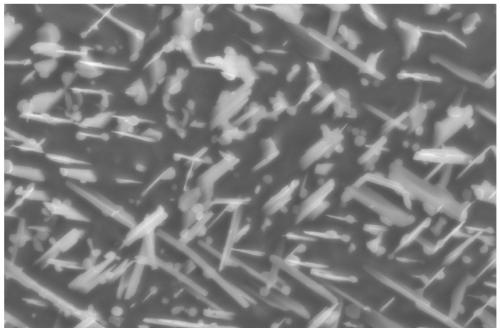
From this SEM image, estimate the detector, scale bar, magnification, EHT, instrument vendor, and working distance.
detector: InLens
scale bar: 200 nm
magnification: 30 K X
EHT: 5 kV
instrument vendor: Zeiss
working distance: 6.3 mm